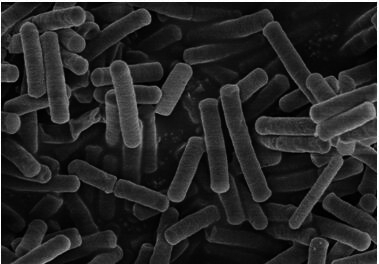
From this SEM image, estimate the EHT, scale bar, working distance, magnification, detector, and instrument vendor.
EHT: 3 kV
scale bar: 5000 nm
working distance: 8.7 mm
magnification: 10 K X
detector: SE(M)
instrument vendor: Hitachi